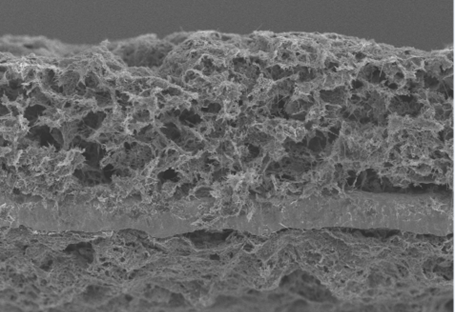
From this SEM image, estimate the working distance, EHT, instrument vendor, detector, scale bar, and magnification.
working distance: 8.8 mm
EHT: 5 kV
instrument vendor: Hitachi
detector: SE(UL)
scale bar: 50000 nm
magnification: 1 K X